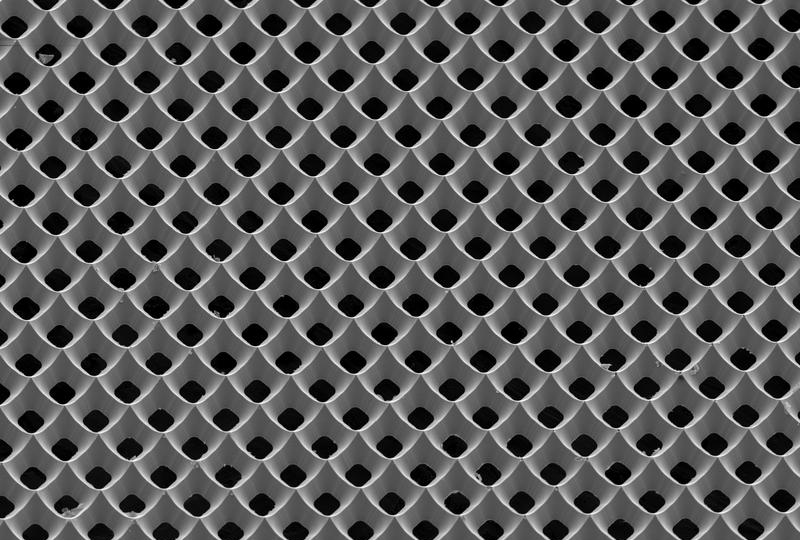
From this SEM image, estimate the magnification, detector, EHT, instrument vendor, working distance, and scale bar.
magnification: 0.25 K X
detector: SEI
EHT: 10 kV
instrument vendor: JEOL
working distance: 31 mm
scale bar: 100000 nm